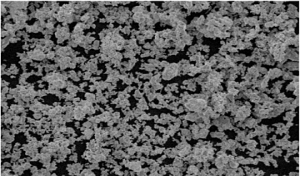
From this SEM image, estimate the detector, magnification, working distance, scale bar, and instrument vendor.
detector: SE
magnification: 2.9 K X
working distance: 11.7 mm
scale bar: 20000 nm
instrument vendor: Tescan